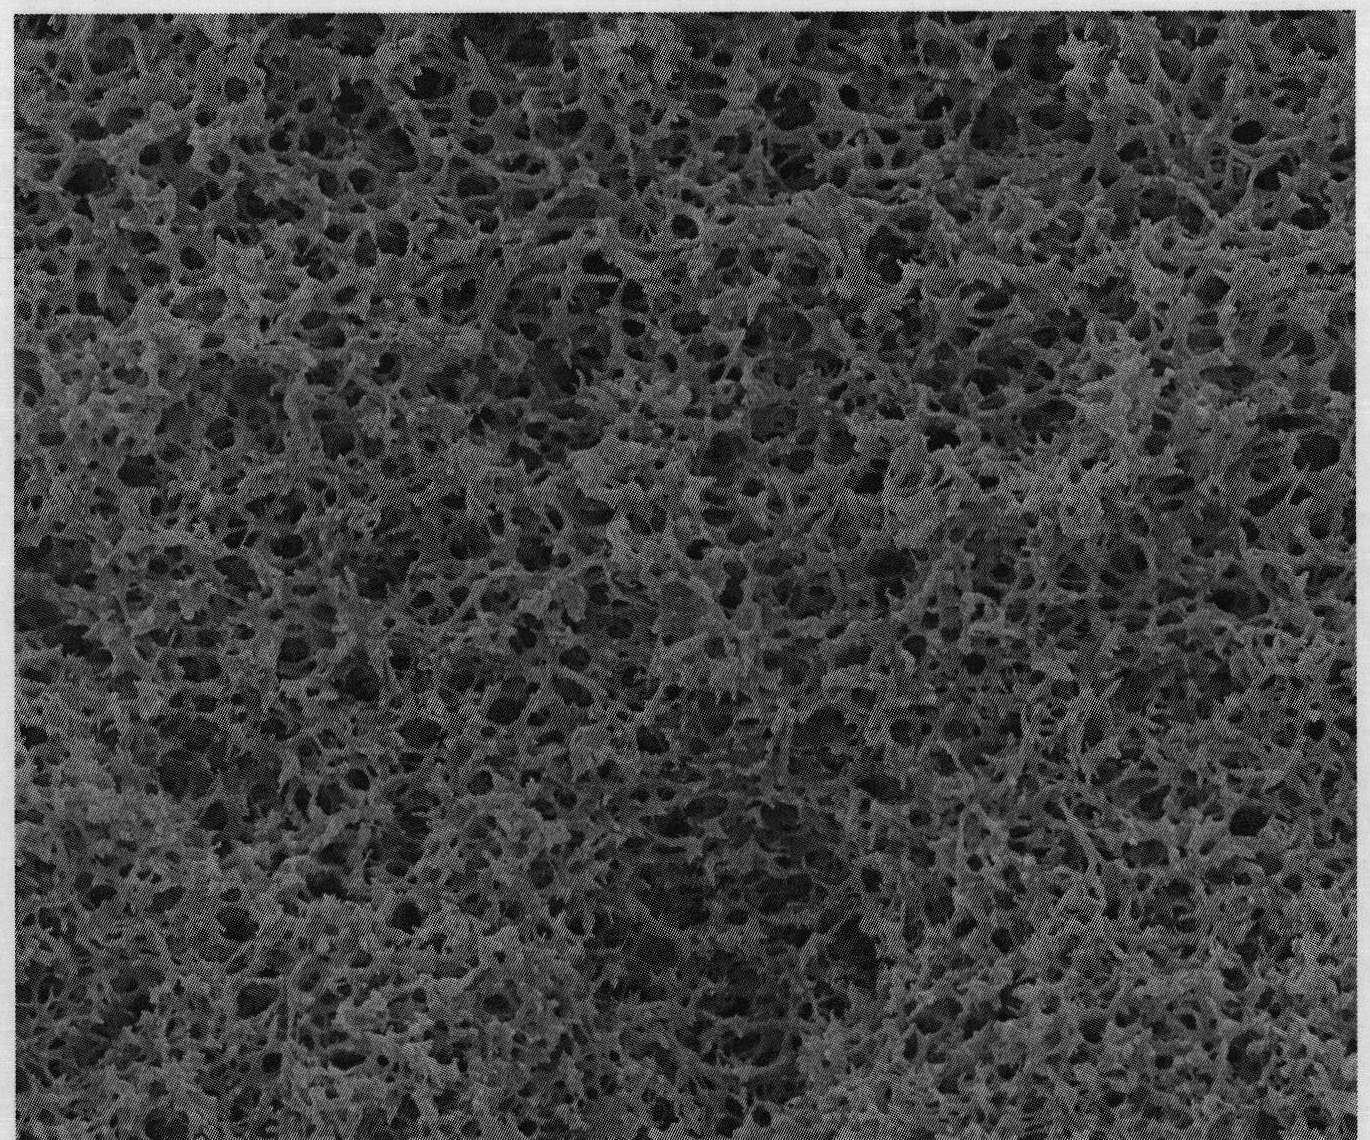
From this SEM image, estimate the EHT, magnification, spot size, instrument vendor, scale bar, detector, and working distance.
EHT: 15 kV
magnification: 10 K X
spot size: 4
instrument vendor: FEI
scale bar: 5000 nm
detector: ETD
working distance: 10 mm